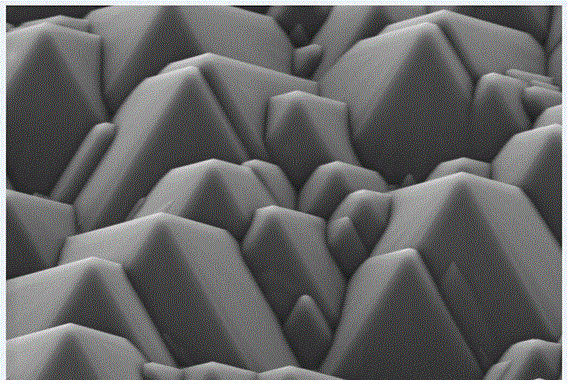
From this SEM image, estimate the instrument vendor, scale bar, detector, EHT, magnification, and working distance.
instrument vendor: Zeiss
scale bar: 1000 nm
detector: SE2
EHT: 5 kV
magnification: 10 K X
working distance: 8.6 mm